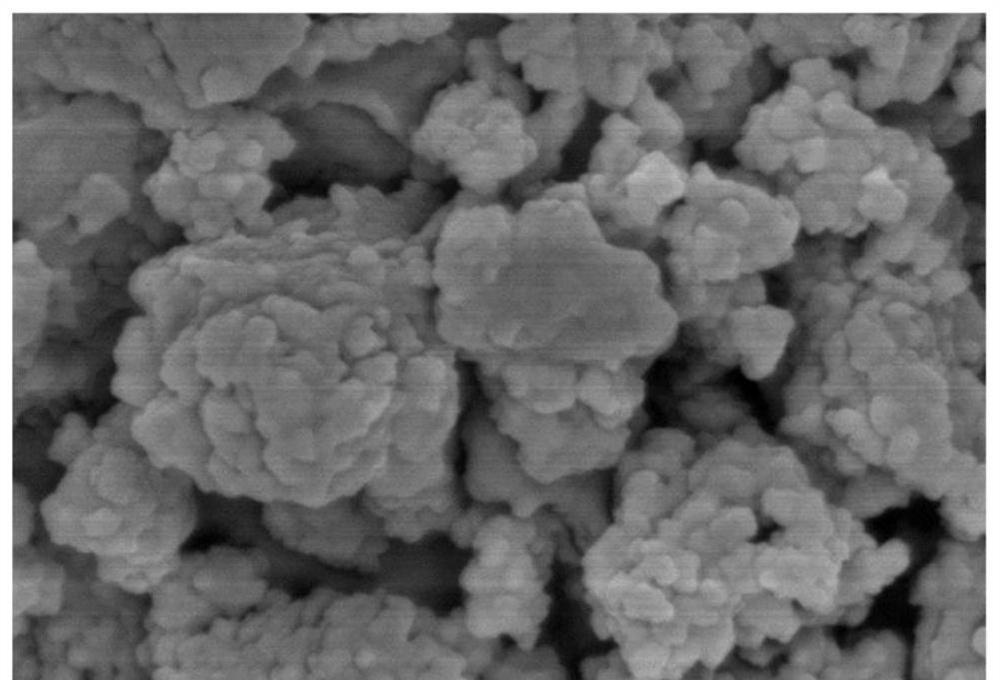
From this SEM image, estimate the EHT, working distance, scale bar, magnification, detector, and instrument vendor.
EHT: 5 kV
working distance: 7.8 mm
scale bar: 500 nm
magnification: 100 K X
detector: SE(U)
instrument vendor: Hitachi